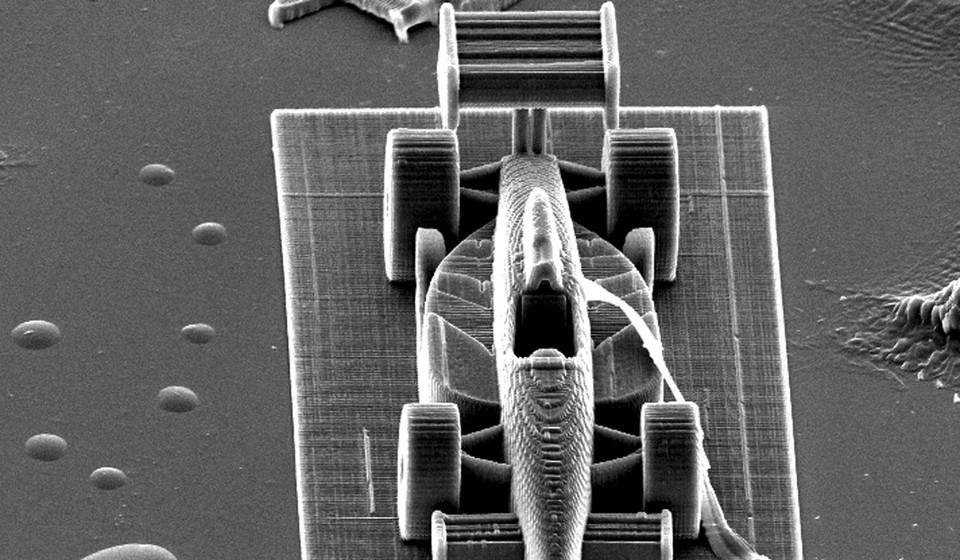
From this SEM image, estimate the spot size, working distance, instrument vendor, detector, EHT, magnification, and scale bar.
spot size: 4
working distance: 27.2 mm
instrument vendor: FEI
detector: SE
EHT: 20 kV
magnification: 0.297 K X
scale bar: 100000 nm